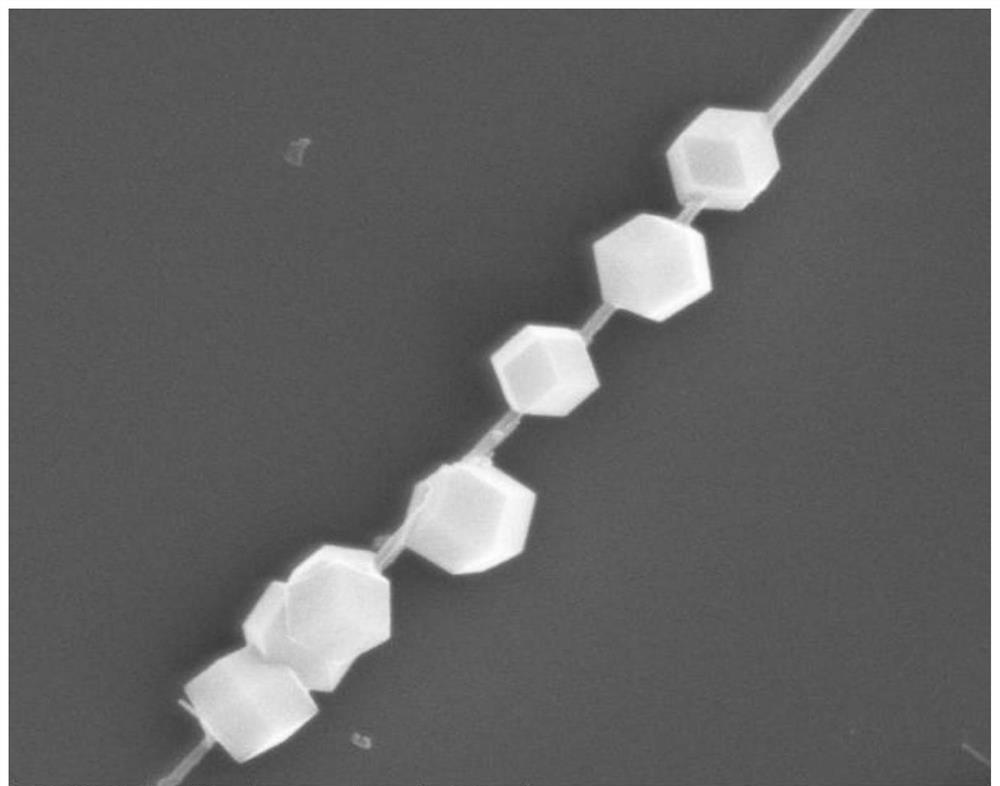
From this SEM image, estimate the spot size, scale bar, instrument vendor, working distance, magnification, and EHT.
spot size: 3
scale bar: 2000 nm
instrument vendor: FEI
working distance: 16.8 mm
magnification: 50 K X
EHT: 10 kV